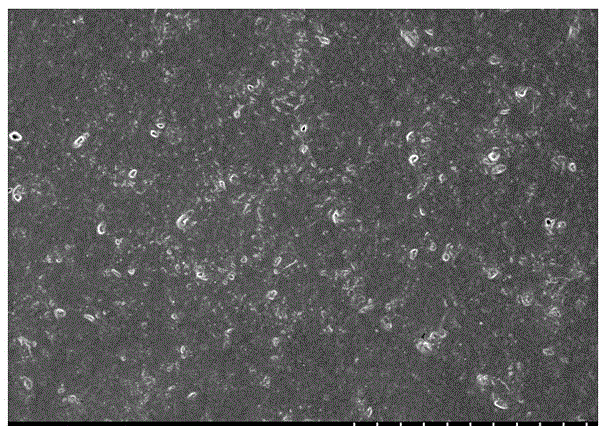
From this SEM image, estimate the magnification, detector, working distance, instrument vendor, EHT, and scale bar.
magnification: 1 K X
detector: SE(M)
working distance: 8.7 mm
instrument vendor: Hitachi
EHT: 3 kV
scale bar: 50000 nm